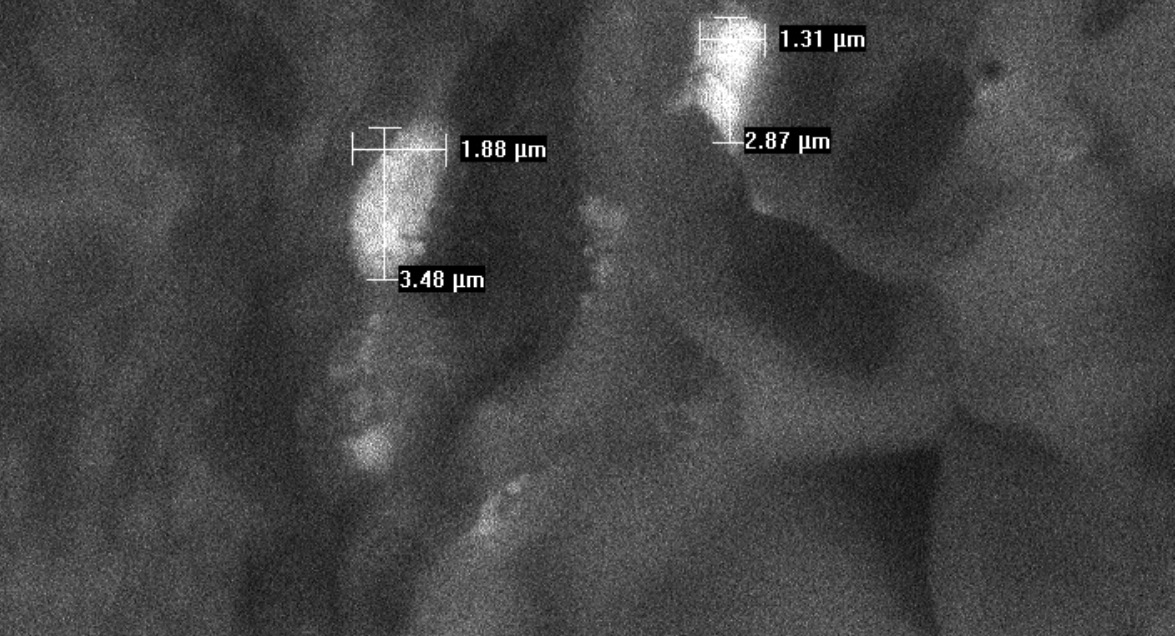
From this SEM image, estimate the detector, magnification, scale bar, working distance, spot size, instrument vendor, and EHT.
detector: BSE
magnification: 4.947 K X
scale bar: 5000 nm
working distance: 11.4 mm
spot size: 5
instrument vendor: FEI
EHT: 20 kV